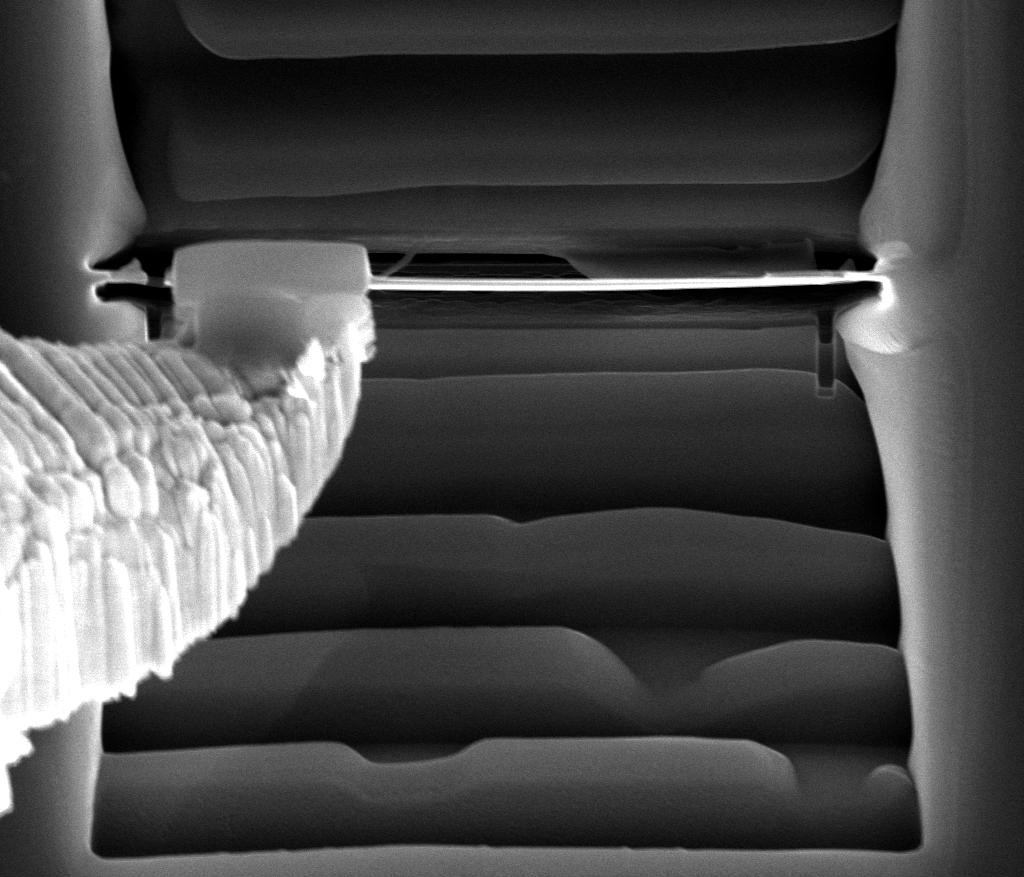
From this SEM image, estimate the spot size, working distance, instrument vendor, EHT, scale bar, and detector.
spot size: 4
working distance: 10 mm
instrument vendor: FEI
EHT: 20 kV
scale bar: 10000 nm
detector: ETD